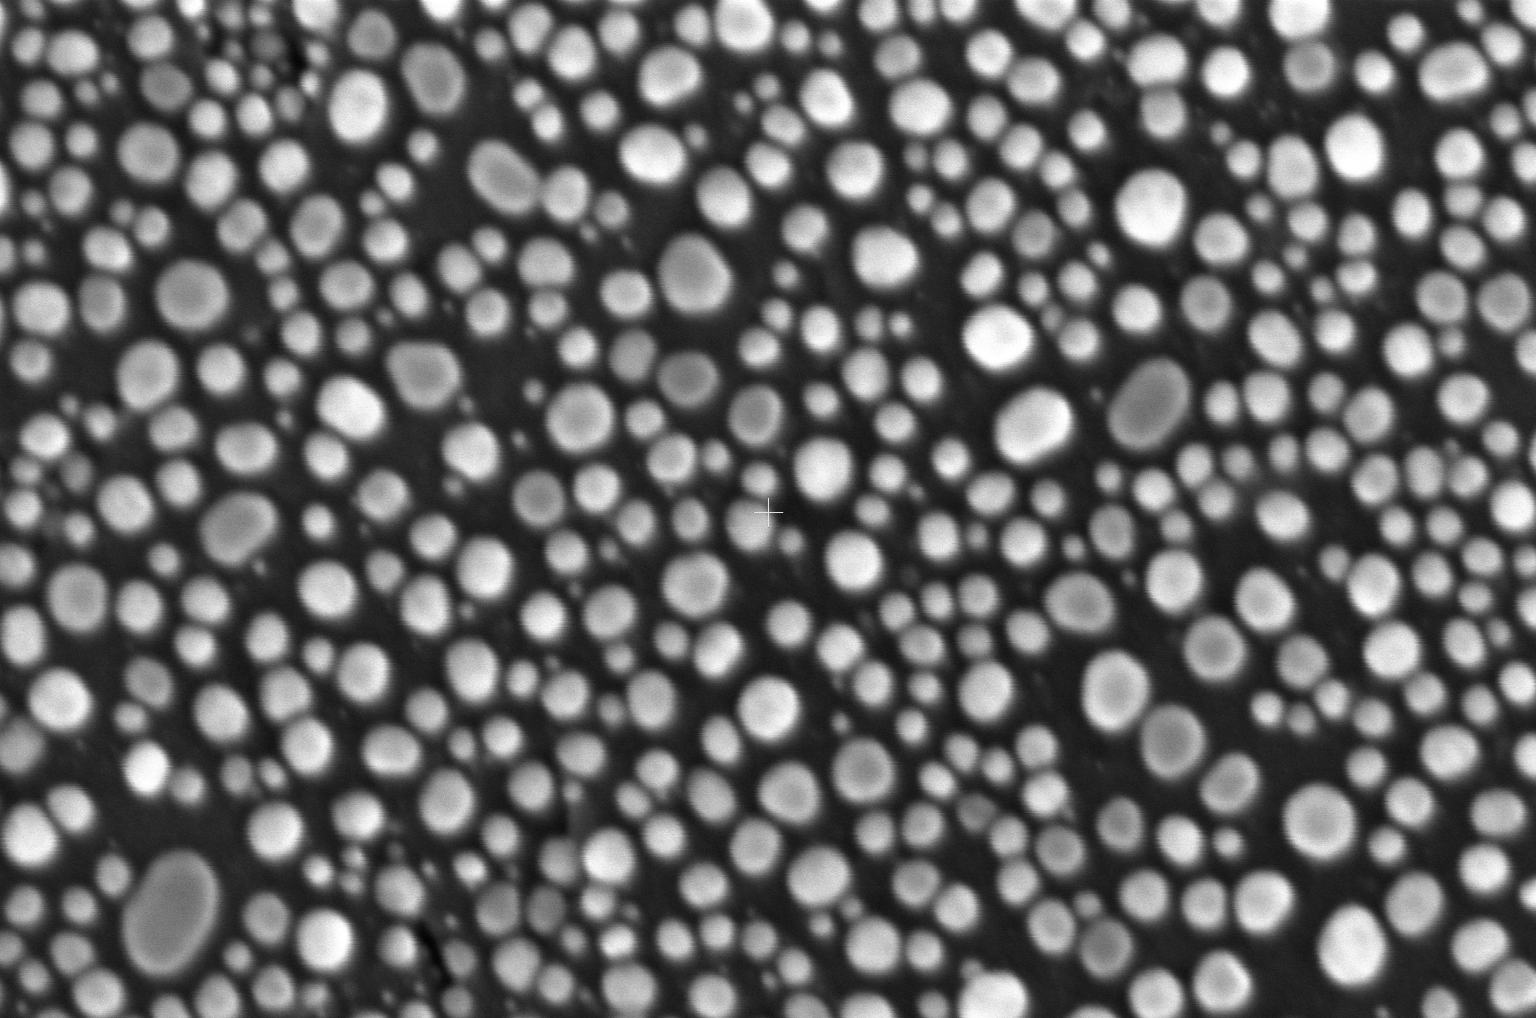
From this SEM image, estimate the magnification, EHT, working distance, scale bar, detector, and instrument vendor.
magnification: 600 K X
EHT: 10 kV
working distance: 4.1 mm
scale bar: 300 nm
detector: TLD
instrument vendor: Thermo